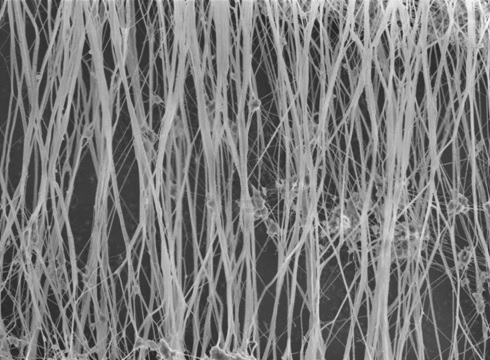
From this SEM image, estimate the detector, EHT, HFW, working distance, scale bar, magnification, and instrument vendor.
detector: TLD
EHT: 5 kV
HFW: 41.4 µm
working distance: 4.1 mm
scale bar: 20000 nm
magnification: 5 K X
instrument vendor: FEI